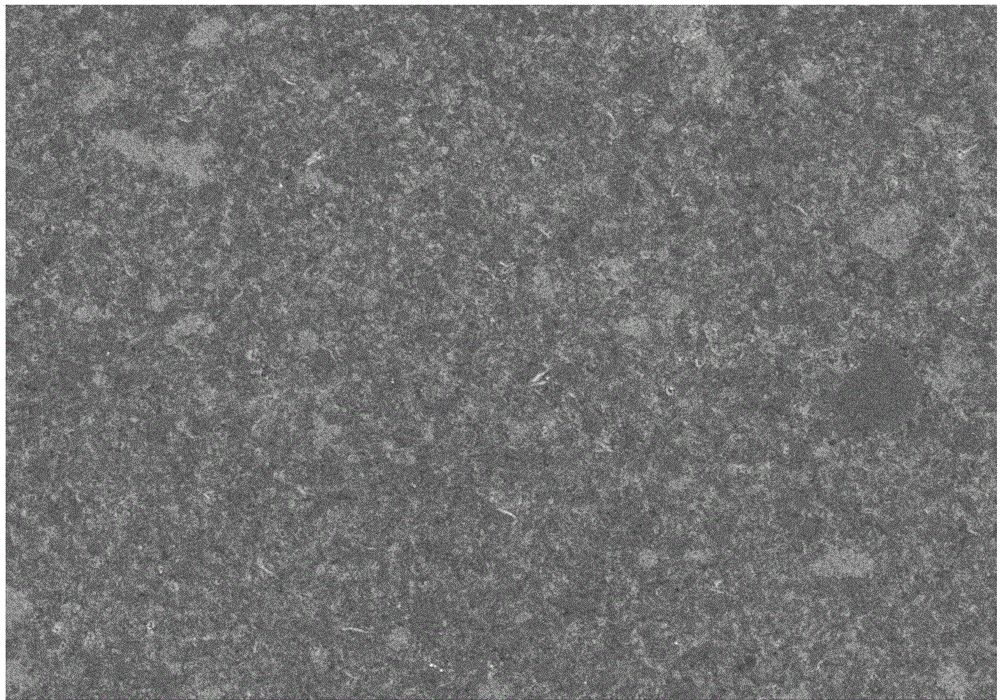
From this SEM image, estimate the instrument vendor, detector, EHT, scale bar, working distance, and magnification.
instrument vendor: Hitachi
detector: SE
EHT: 5 kV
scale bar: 50000 nm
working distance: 10.4 mm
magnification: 1 K X